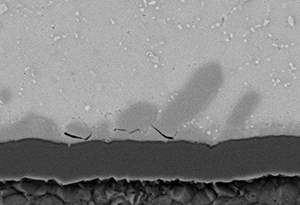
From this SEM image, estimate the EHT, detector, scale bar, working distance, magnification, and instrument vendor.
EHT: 15 kV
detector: BSE3D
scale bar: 10000 nm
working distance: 6.9 mm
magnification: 5 K X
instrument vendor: Hitachi